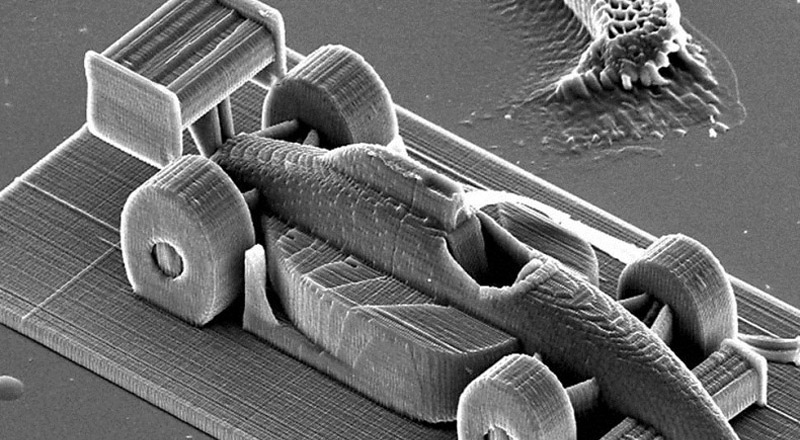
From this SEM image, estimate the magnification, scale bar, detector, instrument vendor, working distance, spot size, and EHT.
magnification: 0.364 K X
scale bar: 100000 nm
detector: SE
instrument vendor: FEI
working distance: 27.2 mm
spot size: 4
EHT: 20 kV